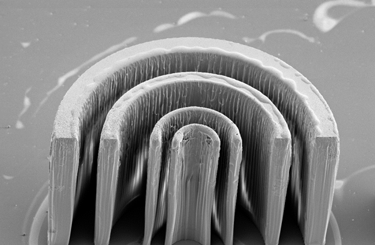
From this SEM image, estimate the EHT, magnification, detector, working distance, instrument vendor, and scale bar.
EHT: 10 kV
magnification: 0.128 K X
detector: SE2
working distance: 21 mm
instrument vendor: Zeiss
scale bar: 100000 nm